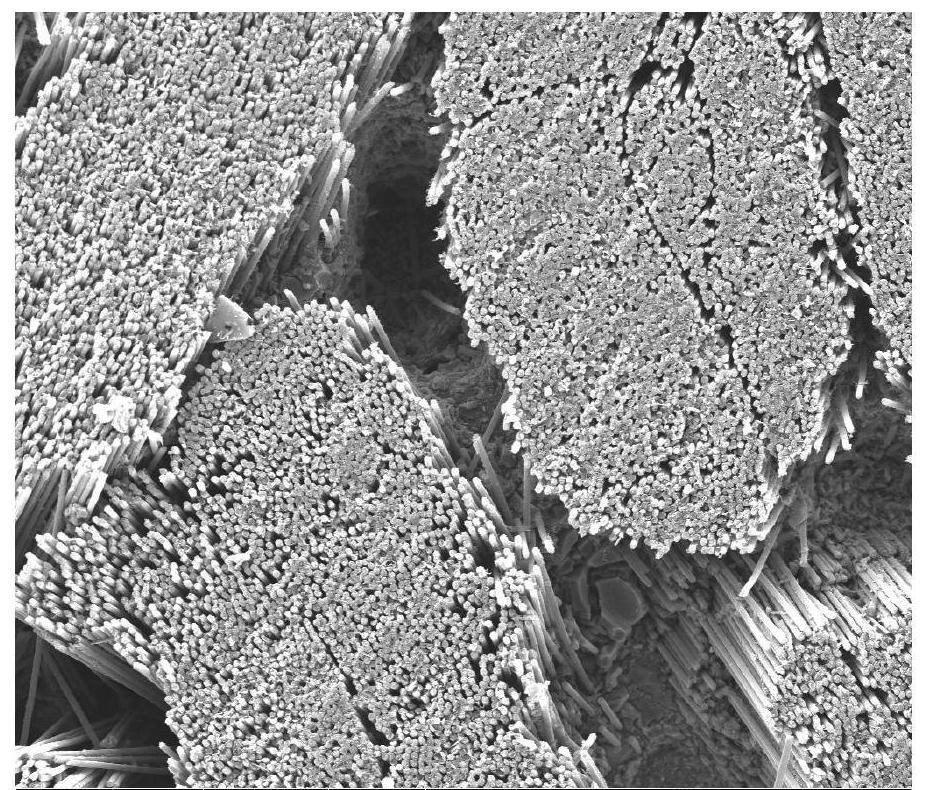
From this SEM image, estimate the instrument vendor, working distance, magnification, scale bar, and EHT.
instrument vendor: FEI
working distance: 14 mm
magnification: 0.3 K X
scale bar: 400000 nm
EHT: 30 kV